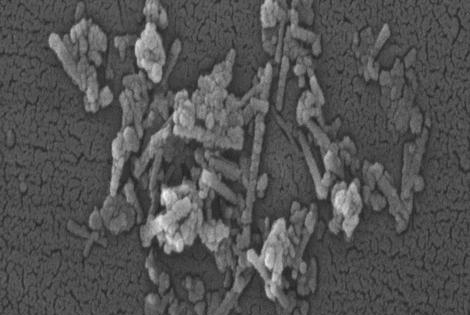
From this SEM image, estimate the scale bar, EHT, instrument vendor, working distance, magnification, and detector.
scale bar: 100 nm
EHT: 15 kV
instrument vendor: Zeiss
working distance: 11.8 mm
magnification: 100 K X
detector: SE2